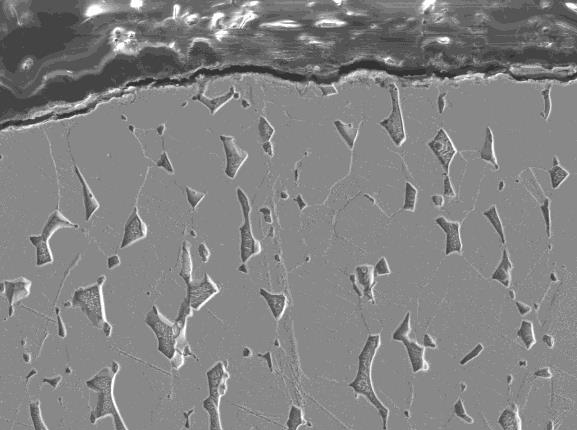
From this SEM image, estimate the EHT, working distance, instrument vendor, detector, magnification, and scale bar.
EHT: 15 kV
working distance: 8.9 mm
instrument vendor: JEOL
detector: LEI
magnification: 1 K X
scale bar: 10000 nm